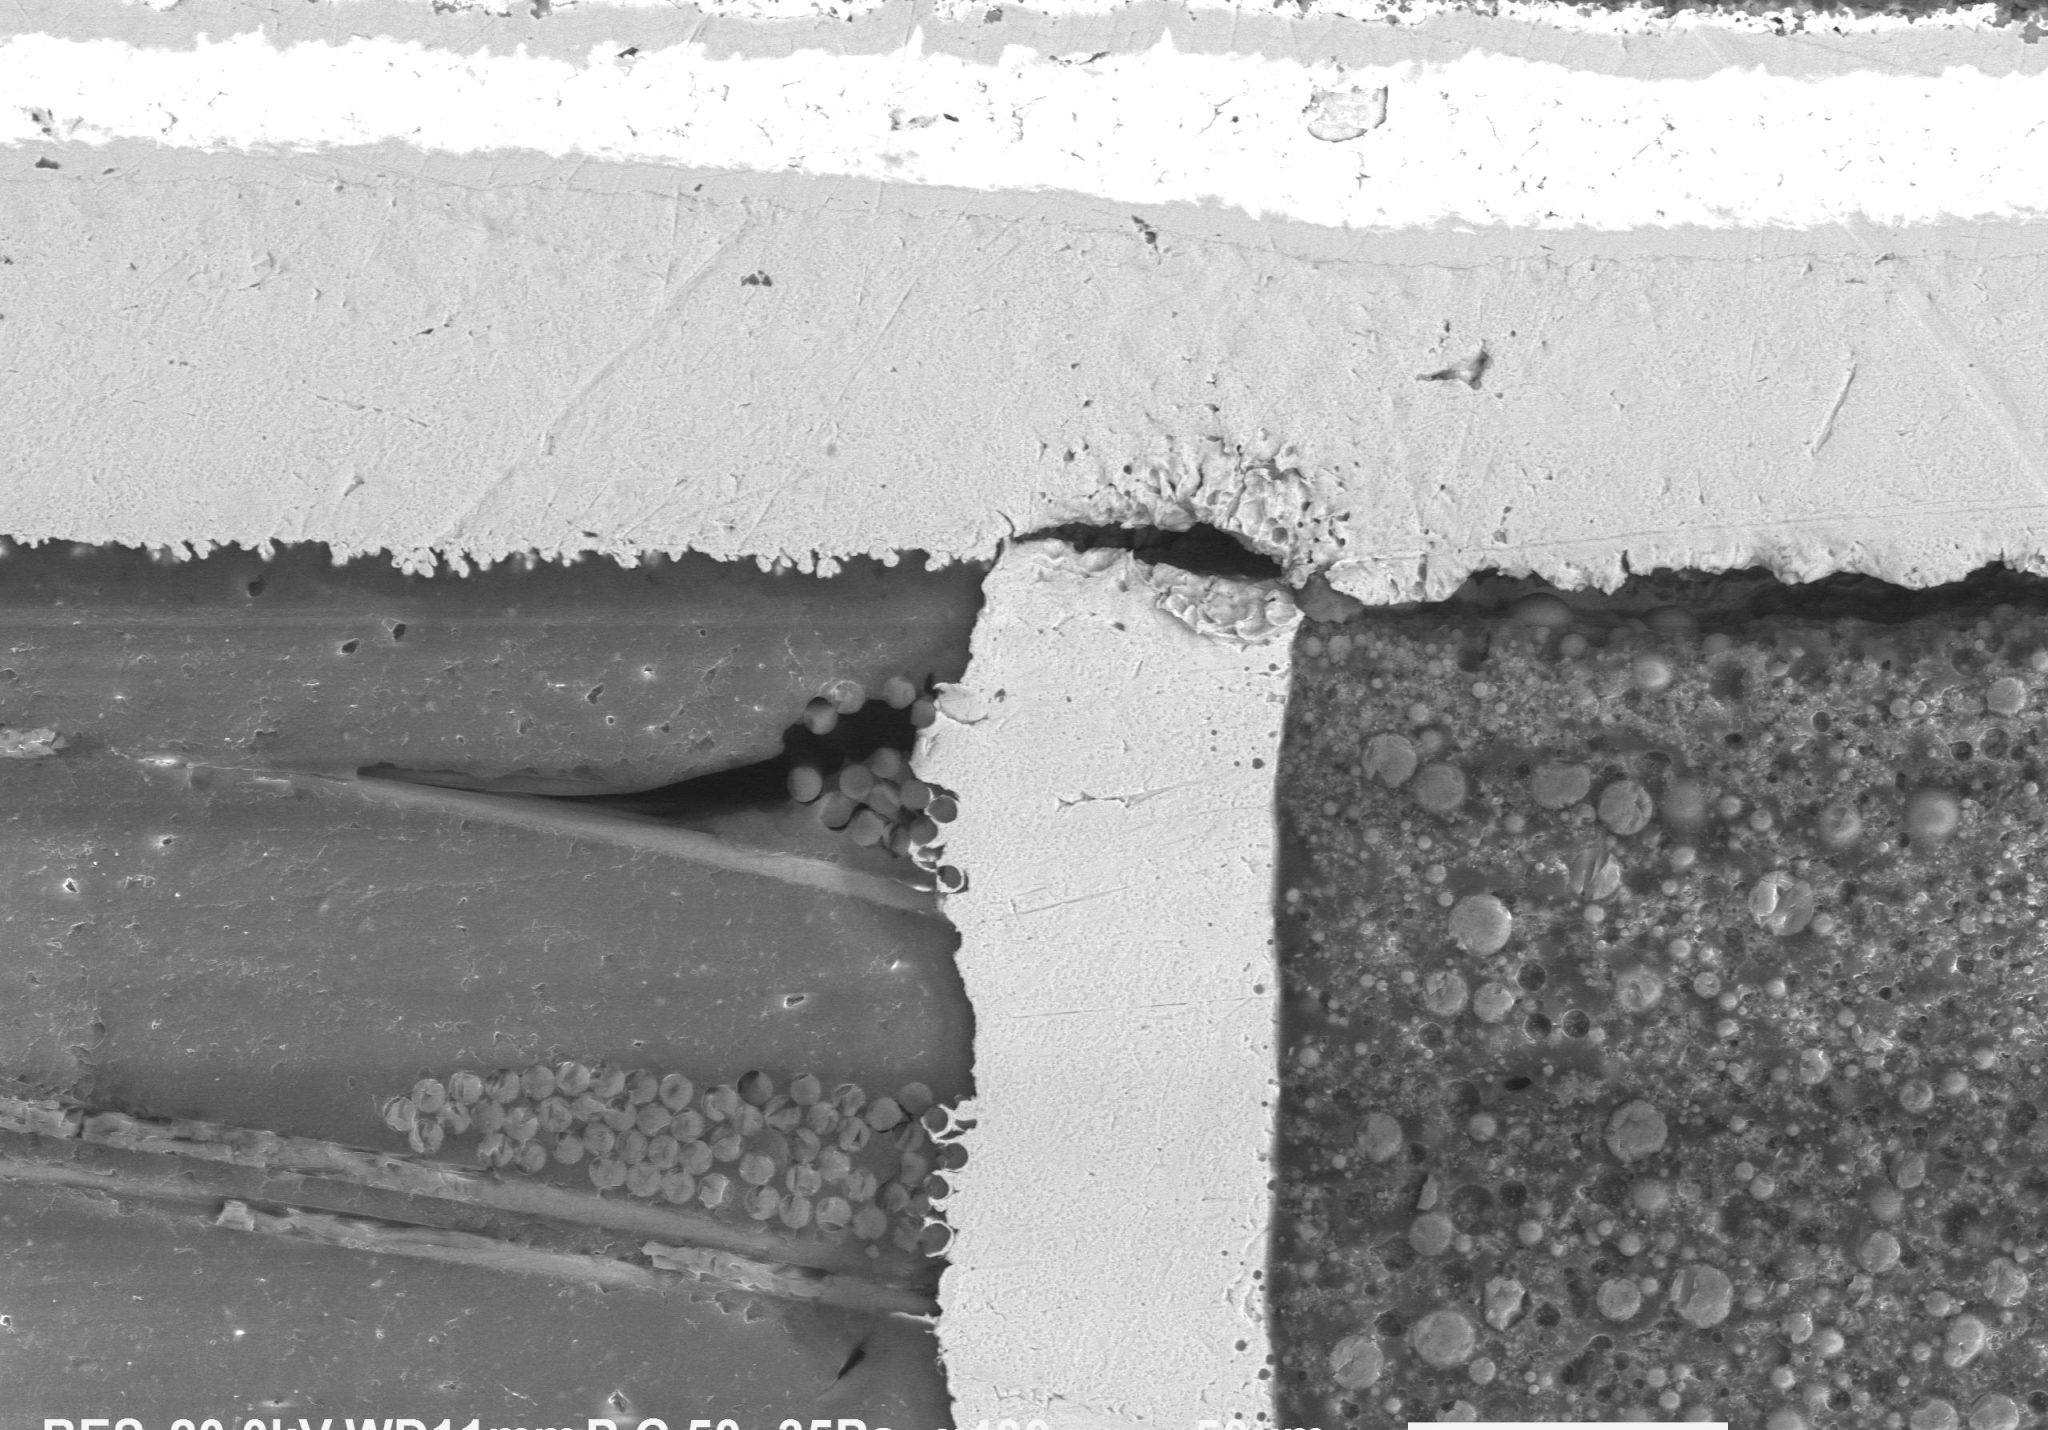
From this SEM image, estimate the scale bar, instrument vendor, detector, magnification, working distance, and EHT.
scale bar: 50000 nm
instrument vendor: JEOL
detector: BES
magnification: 0.4 K X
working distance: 11 mm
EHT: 20 kV